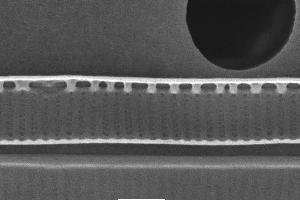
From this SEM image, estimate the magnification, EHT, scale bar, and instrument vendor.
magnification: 20 K X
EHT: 10 kV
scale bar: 1000 nm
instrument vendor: JEOL